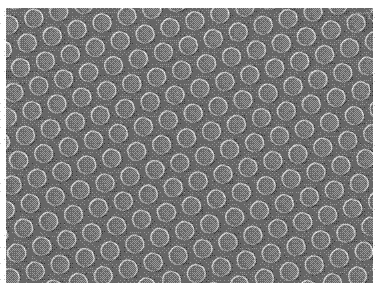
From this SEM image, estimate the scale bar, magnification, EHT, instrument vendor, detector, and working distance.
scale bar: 10000 nm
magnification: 2 K X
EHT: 5 kV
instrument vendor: JEOL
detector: SEI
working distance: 4 mm